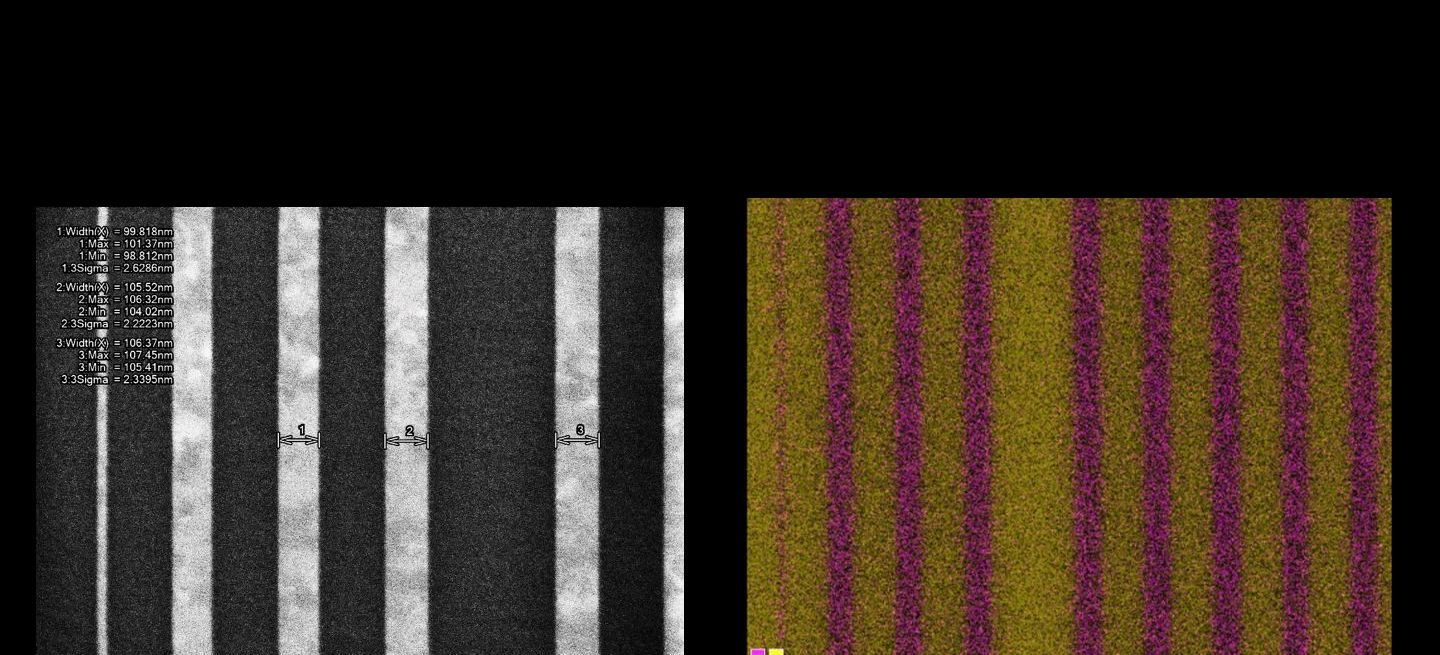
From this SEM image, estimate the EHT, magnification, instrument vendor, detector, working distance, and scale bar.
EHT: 0.8 kV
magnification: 80 K X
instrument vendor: Hitachi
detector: LA100(U)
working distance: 1.8 mm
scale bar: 500 nm